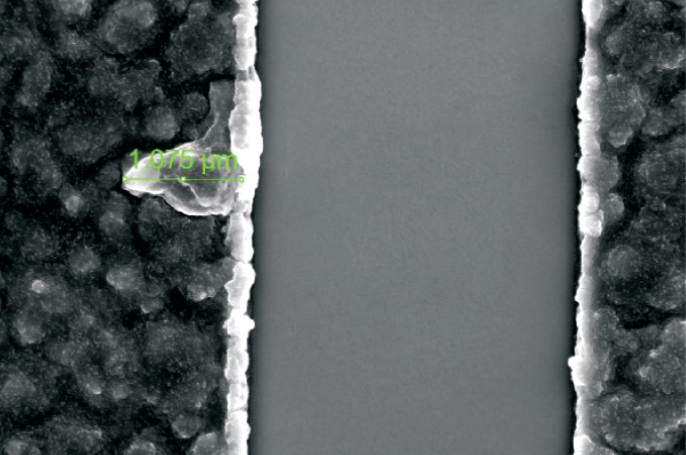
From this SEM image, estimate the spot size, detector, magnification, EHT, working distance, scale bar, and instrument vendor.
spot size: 3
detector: SE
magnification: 20 K X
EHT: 10 kV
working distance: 5 mm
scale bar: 2000 nm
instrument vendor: FEI